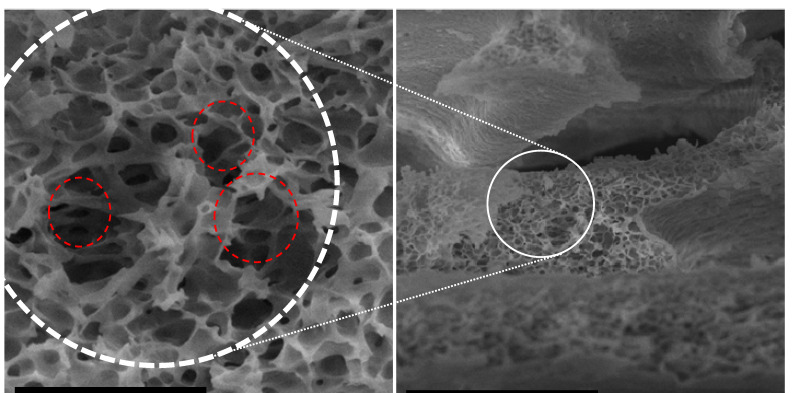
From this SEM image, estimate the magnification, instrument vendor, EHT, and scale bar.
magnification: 20 K X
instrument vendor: JEOL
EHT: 7 kV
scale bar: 1000 nm